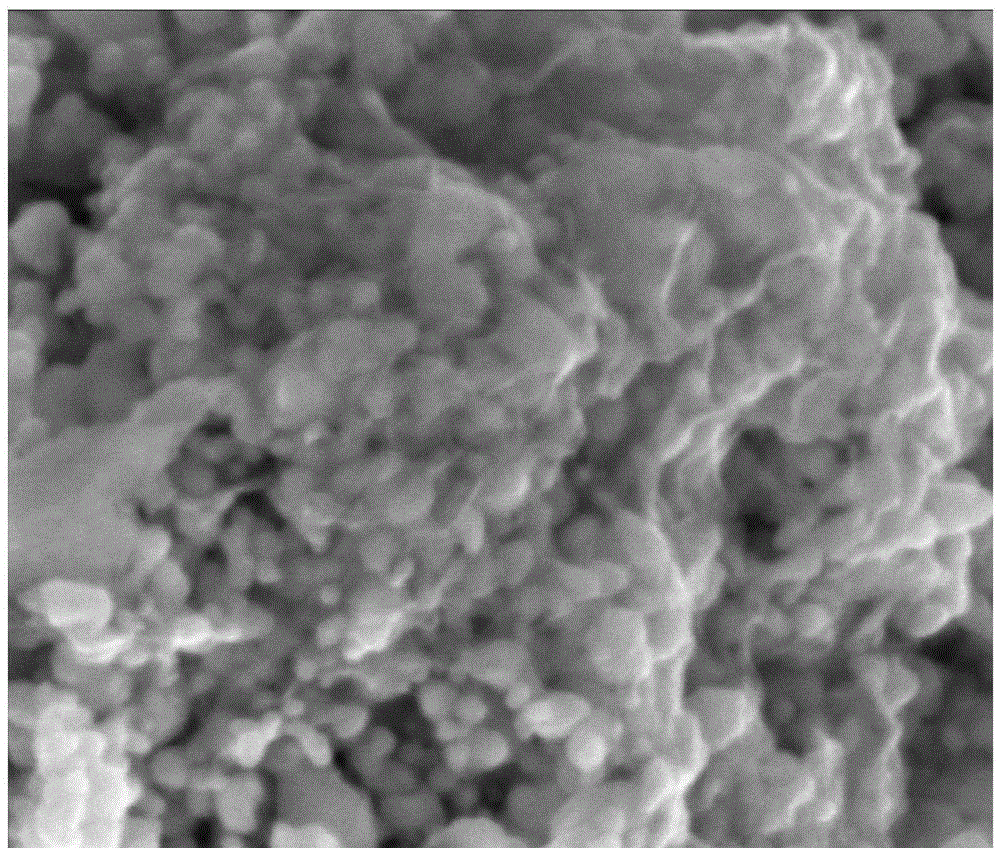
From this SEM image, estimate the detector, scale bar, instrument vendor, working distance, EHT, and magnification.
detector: ETD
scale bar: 500 nm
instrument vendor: FEI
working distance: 5.6 mm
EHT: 10 kV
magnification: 160 K X